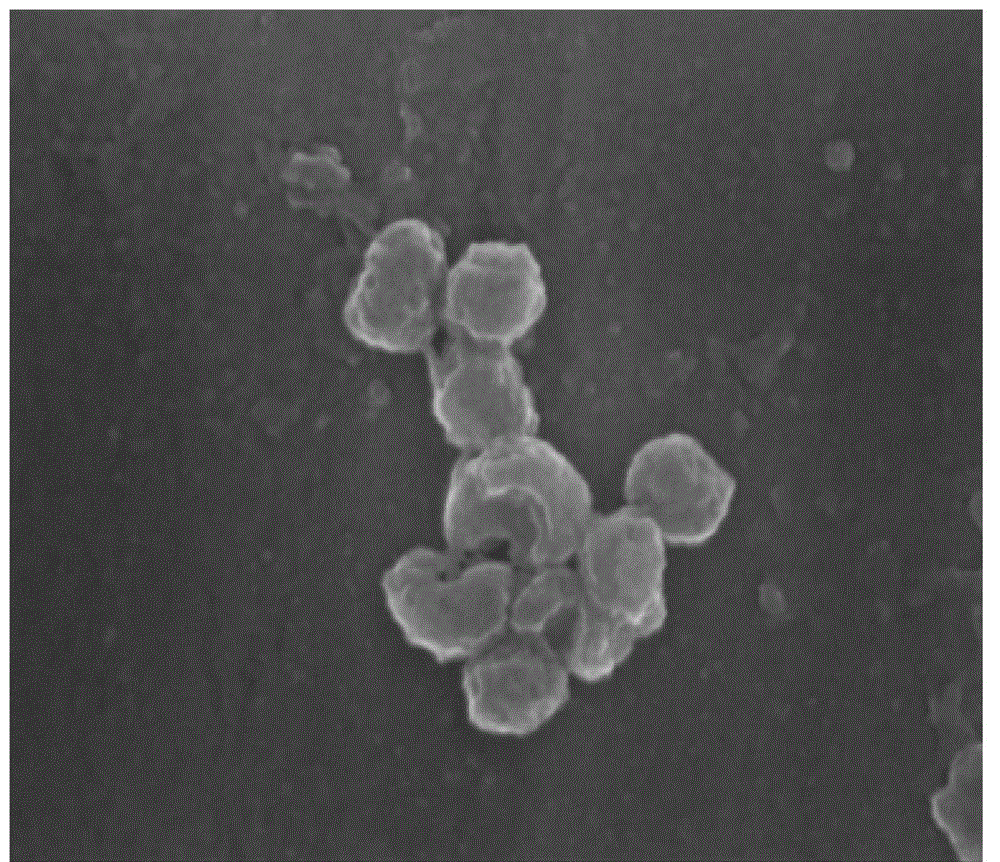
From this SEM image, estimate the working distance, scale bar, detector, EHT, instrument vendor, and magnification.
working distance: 5.8 mm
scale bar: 200 nm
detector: TLD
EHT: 10 kV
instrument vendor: FEI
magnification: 200 K X